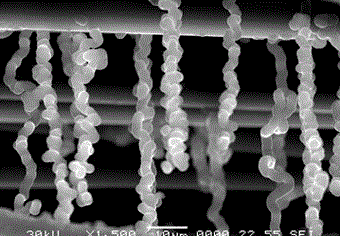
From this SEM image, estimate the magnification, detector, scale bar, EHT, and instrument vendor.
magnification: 1.5 K X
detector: SEI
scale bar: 10000 nm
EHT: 30 kV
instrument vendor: JEOL